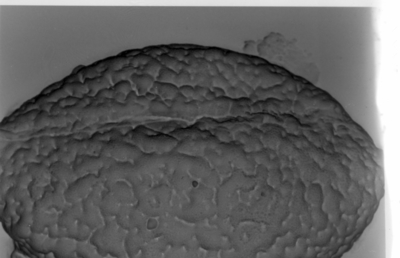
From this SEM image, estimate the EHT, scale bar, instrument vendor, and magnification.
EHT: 15 kV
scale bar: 5000 nm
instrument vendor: JEOL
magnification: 3.5 K X